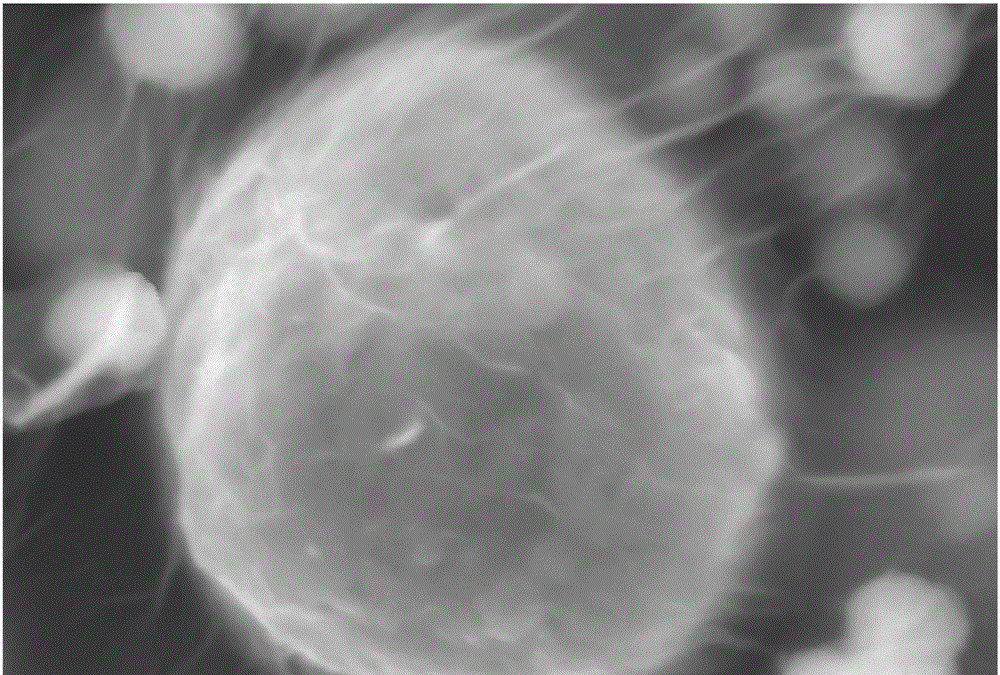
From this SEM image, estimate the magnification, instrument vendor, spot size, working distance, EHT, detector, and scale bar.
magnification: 9 K X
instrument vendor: JEOL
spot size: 50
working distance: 13 mm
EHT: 15 kV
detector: SEI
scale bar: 2000 nm